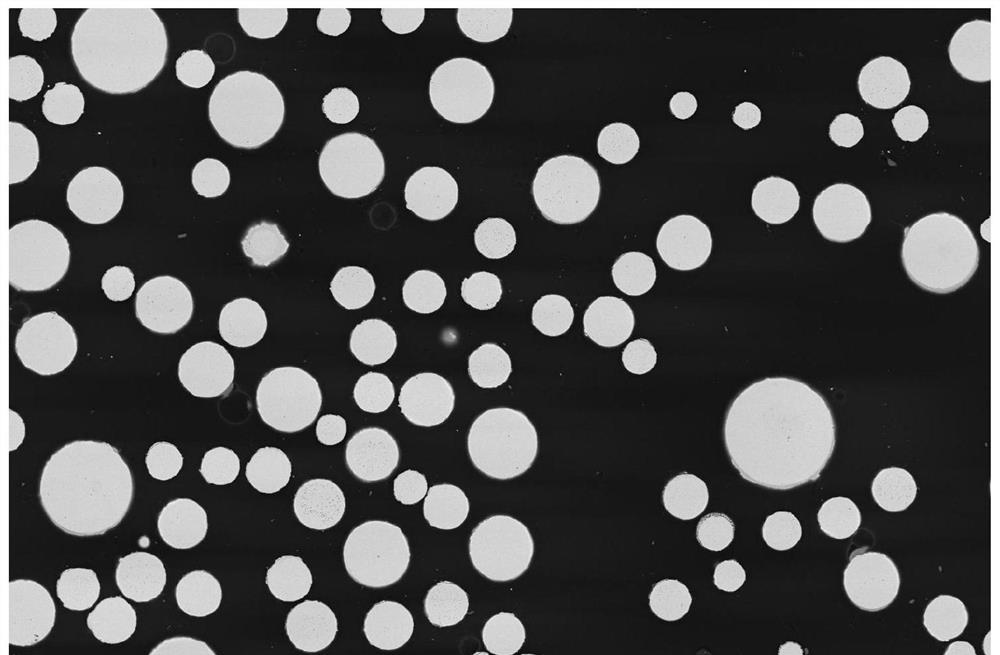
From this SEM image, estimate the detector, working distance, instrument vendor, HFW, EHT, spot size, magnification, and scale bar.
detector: CBS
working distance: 20.7 mm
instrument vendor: FEI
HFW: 1300 µm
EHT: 25 kV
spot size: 5.5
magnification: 0.16 K X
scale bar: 400000 nm